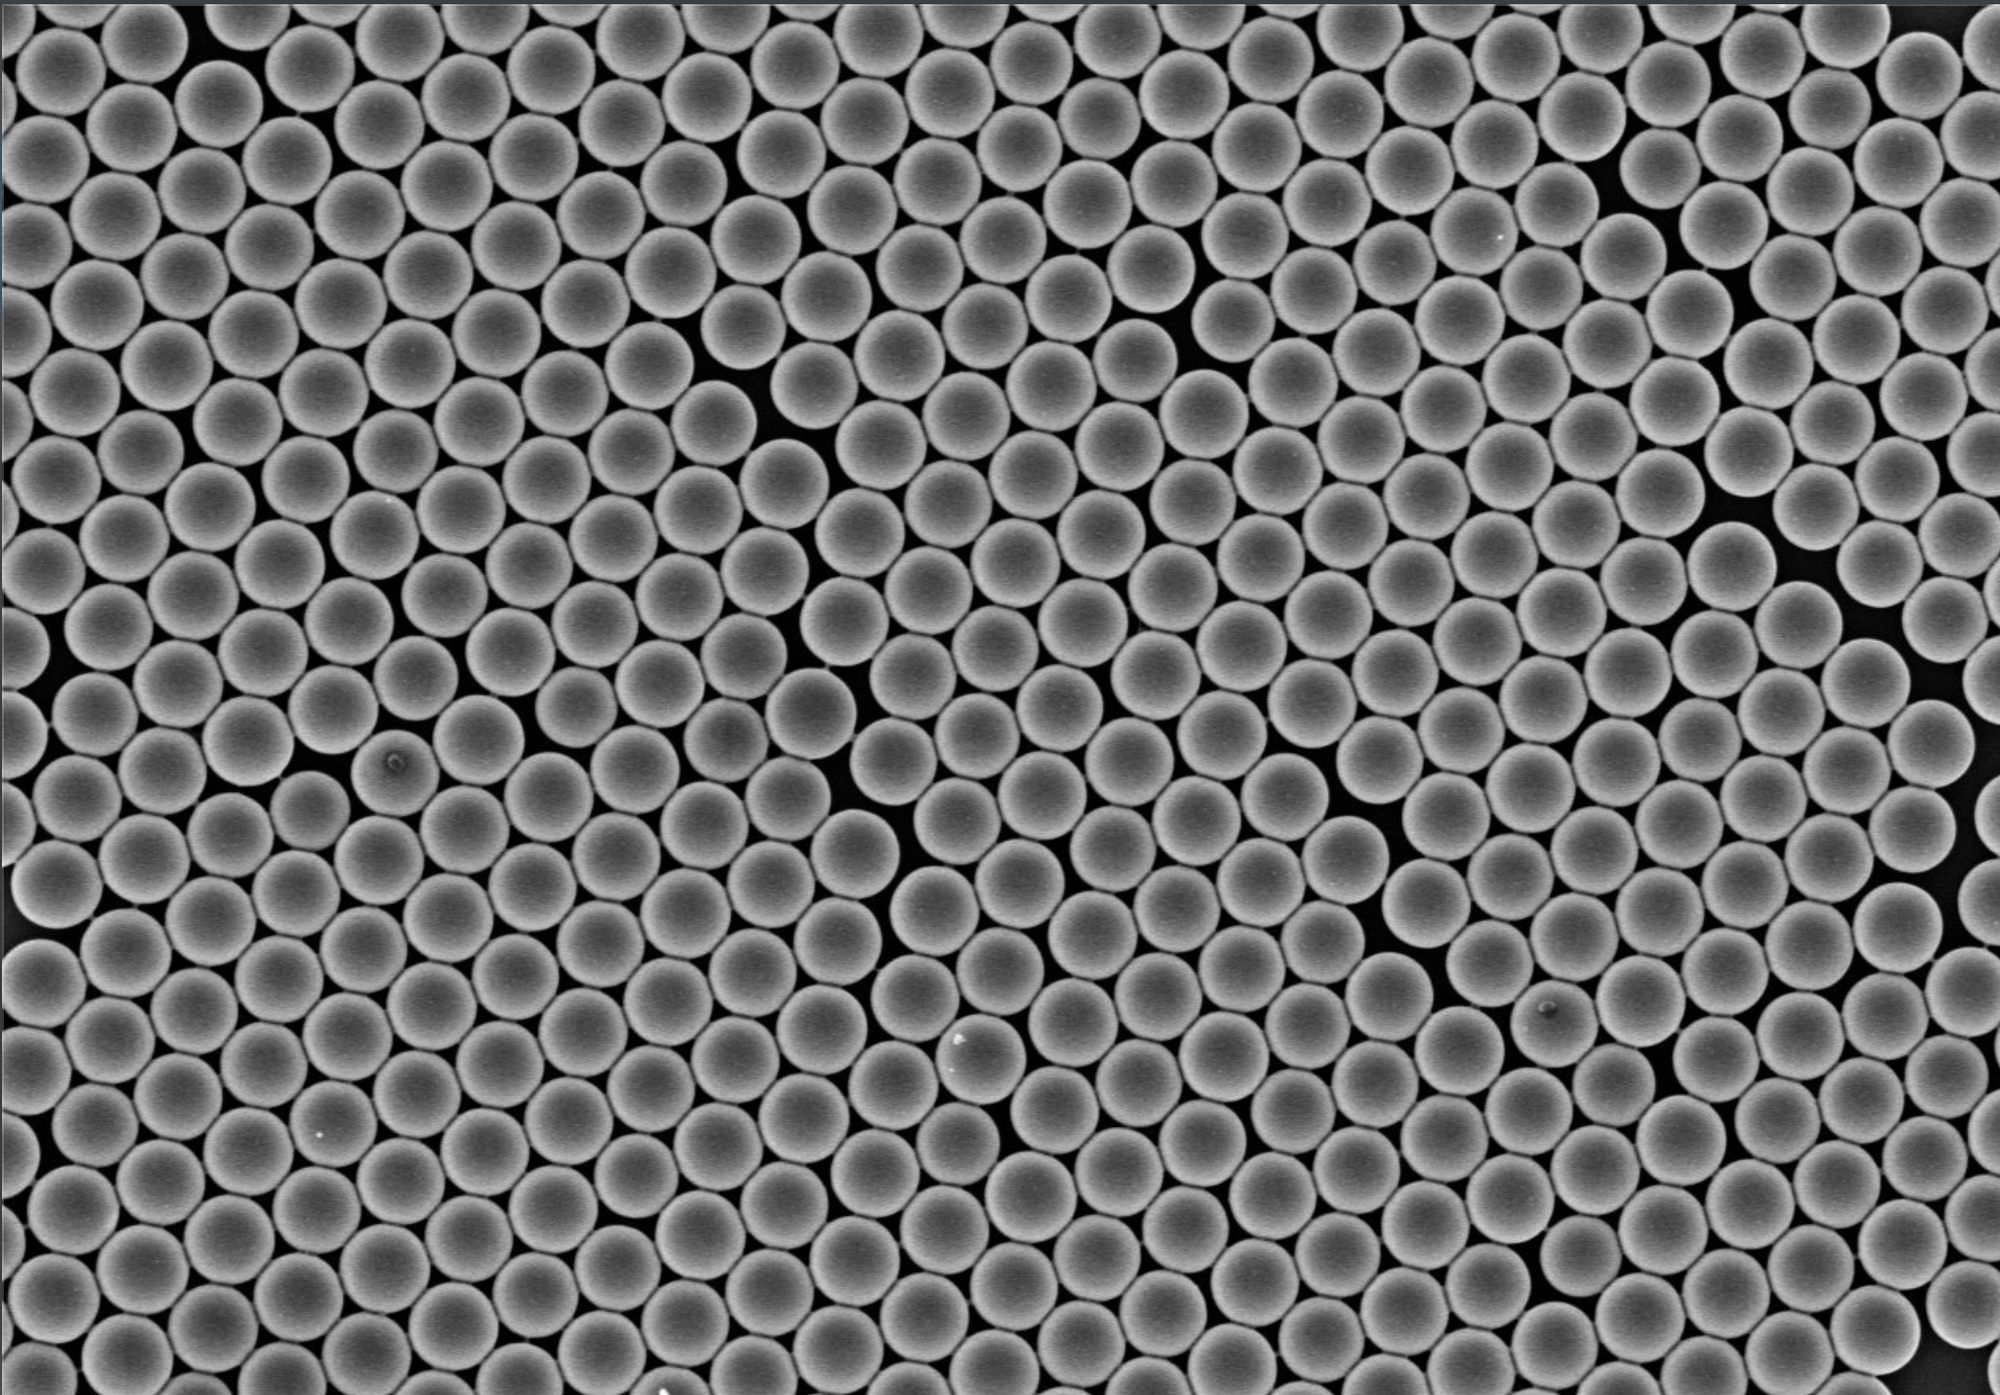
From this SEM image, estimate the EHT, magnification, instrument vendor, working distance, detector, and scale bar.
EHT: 3 kV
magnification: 5 K X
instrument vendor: Hitachi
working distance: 7.5 mm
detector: SE(TUL)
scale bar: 10000 nm